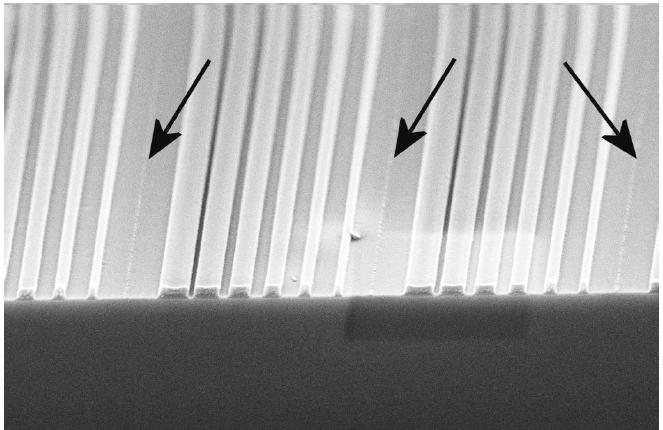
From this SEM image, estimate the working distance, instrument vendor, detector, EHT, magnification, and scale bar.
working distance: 4 mm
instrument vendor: Zeiss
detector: InLens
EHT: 5 kV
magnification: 15.42 K X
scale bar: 1000 nm